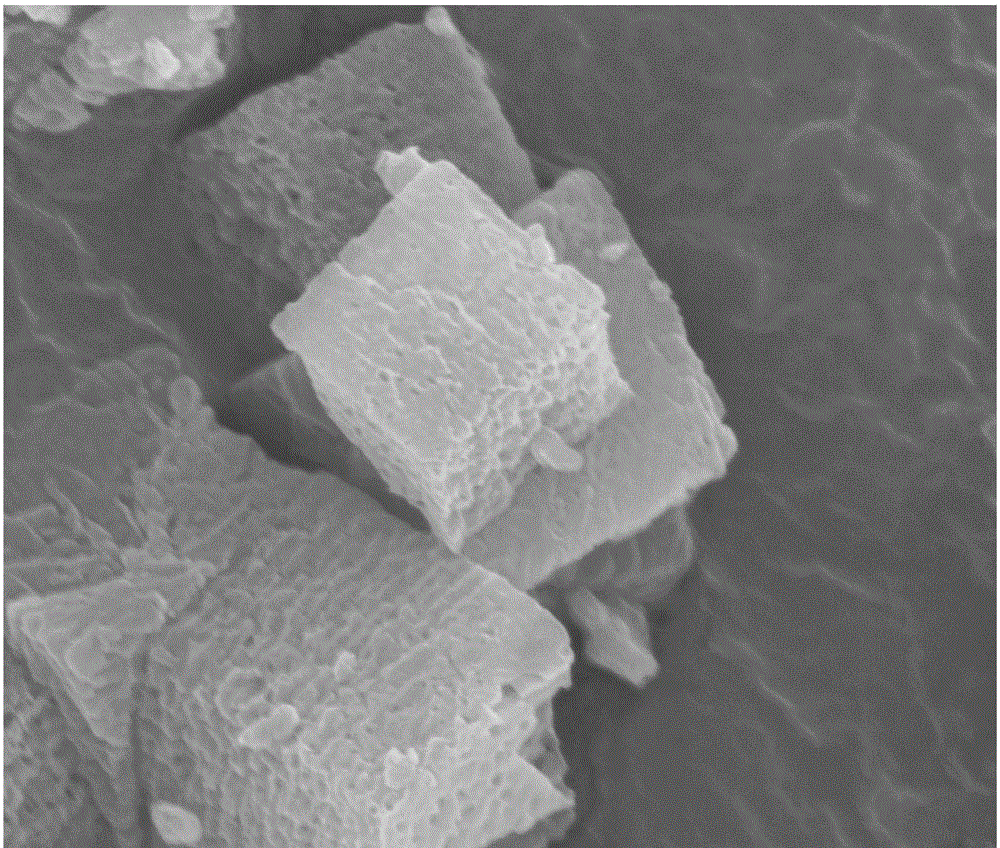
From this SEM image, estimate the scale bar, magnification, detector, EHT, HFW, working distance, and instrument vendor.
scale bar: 1000 nm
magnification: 50 K X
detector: TLD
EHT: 10 kV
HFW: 4.8 µm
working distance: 5.3 mm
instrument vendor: FEI